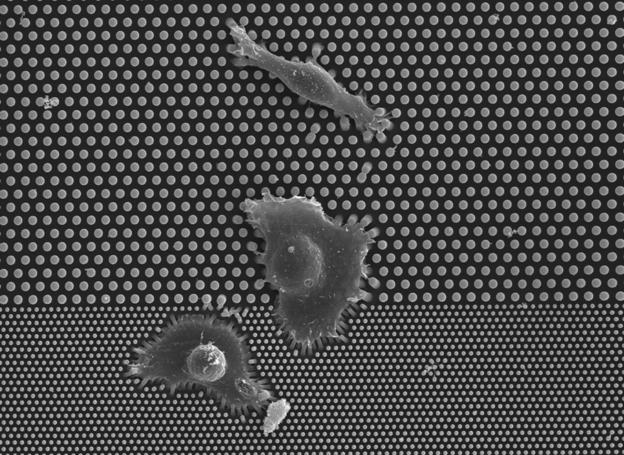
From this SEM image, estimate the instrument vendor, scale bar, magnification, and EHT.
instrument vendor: JEOL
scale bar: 20000 nm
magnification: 0.8 K X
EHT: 15 kV